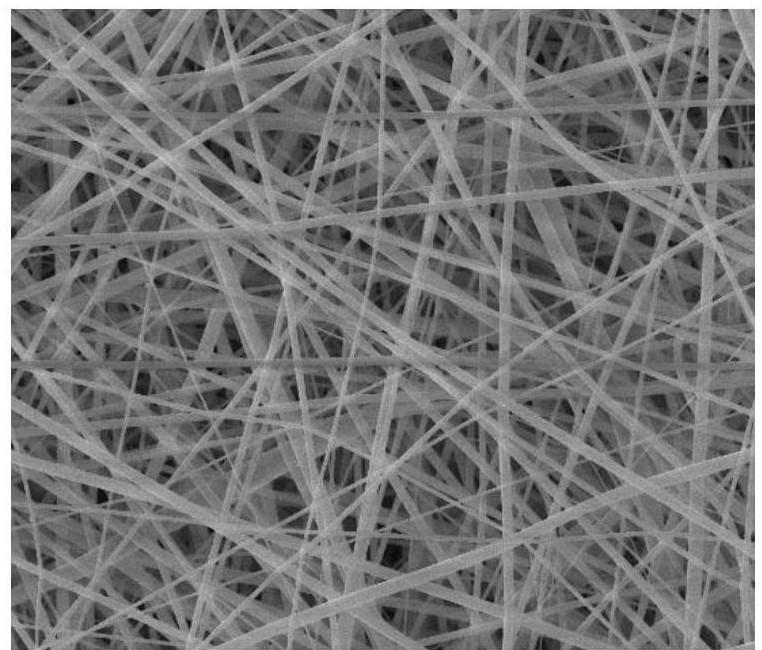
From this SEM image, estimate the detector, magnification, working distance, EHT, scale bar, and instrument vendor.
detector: ETD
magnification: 10 K X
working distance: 11.3 mm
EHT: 10 kV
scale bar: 10000 nm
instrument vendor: FEI